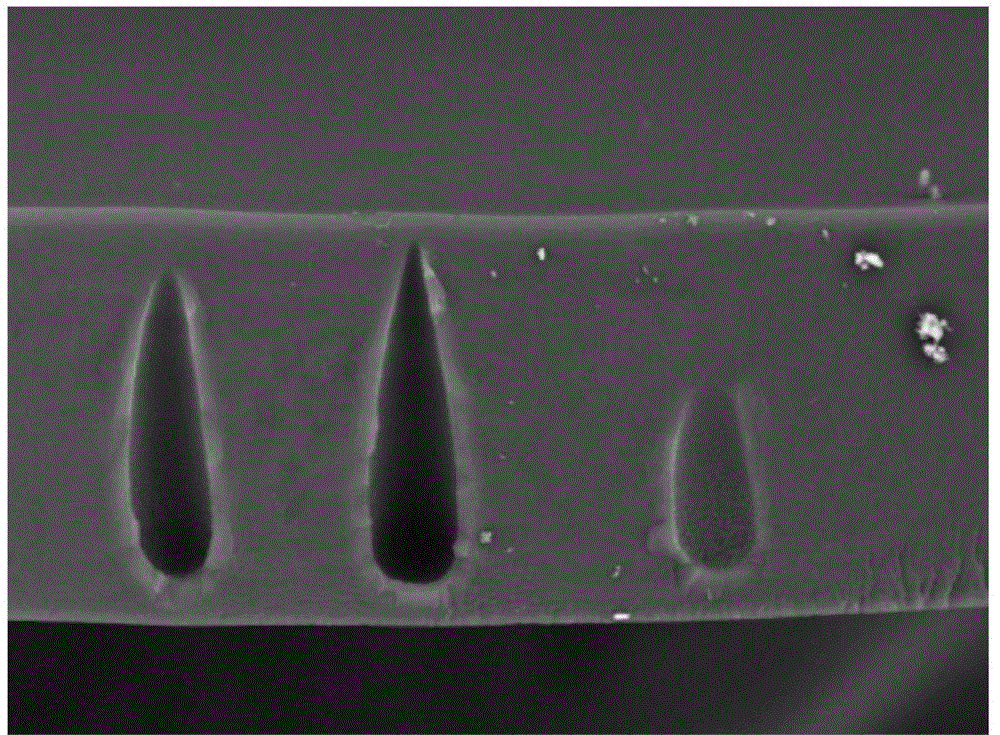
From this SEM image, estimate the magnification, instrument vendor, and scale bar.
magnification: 1 K X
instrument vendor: Hitachi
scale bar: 100000 nm